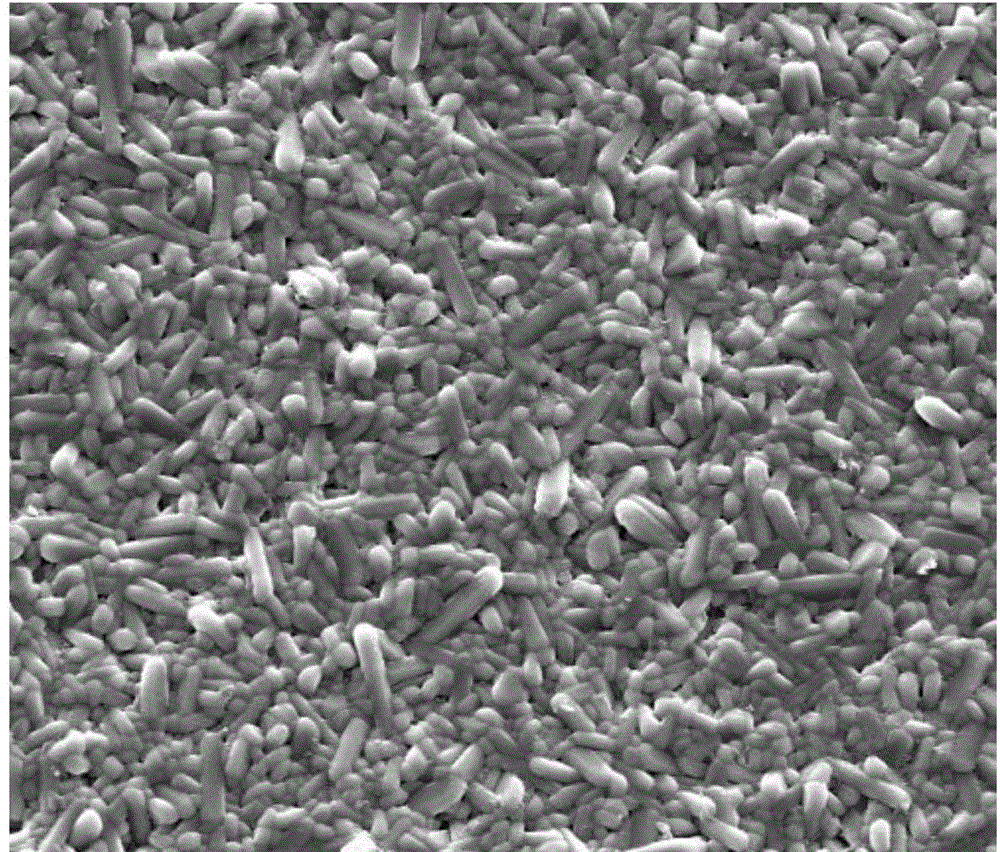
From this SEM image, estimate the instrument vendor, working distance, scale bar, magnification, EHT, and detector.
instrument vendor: FEI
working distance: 9 mm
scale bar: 20000 nm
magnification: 5 K X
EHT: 20 kV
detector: SE